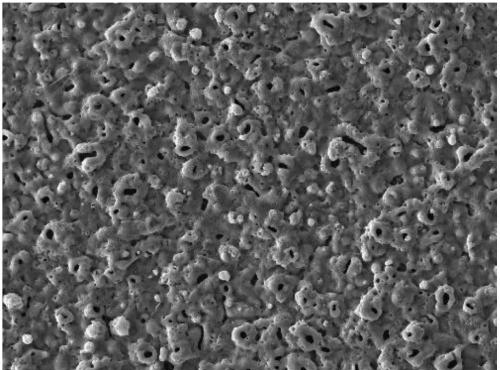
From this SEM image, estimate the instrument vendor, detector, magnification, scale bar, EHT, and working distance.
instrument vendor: Tescan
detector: SE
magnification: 0.5 K X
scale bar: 100000 nm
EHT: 30 kV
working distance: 8.44 mm